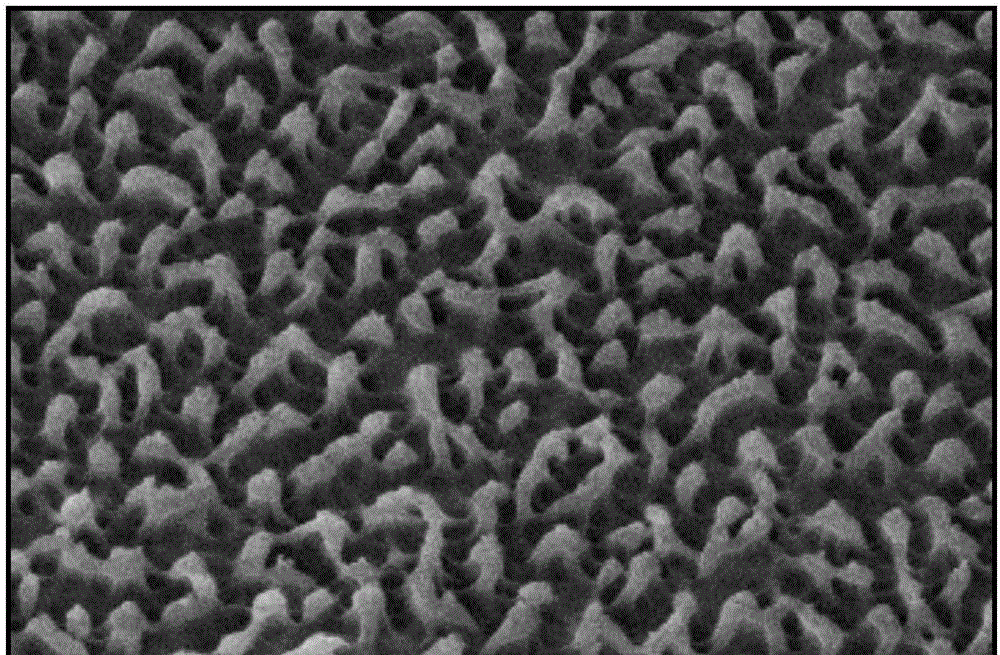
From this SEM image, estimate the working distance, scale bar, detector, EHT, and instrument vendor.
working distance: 9.2 mm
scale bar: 200 nm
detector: InLens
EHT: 5 kV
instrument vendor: Zeiss